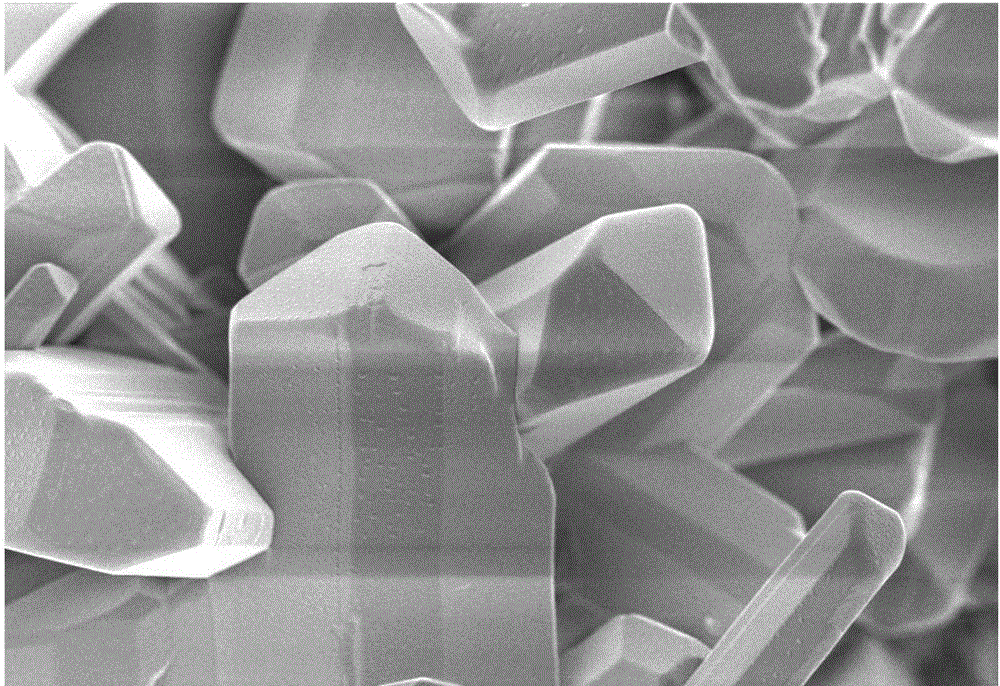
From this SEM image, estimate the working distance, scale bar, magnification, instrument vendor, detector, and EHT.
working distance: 2.7 mm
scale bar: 1000 nm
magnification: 5 K X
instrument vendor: Zeiss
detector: InLens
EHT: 2 kV